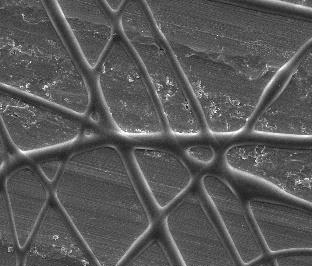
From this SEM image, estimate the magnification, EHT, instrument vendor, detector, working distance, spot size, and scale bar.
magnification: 5 K X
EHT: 5 kV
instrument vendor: FEI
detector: ETD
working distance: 11.1 mm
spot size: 3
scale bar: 10000 nm